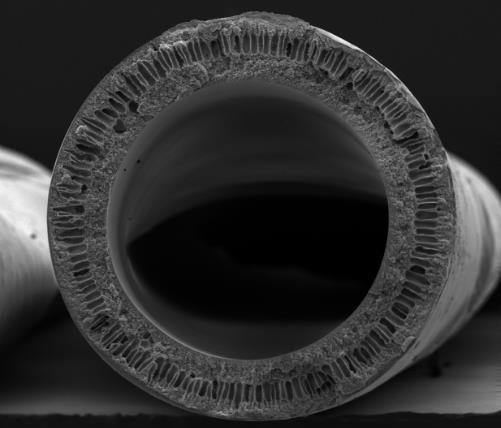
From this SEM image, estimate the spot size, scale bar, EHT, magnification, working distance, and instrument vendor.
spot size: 2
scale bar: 500000 nm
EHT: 10 kV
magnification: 0.17 K X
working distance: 9.9 mm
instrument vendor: FEI